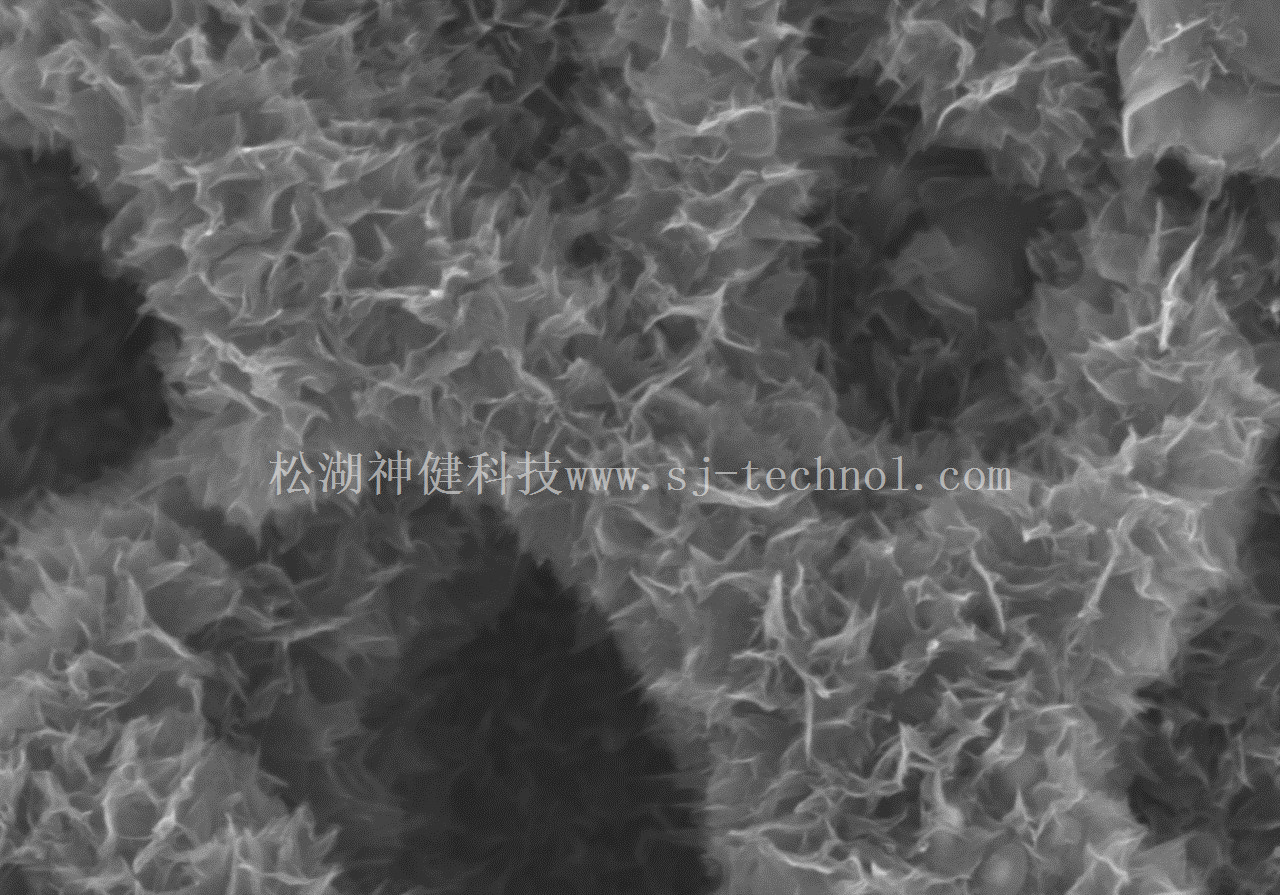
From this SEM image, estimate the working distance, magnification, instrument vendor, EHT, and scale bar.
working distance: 10.8 mm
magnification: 40 K X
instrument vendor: Hitachi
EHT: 5 kV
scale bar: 1000 nm